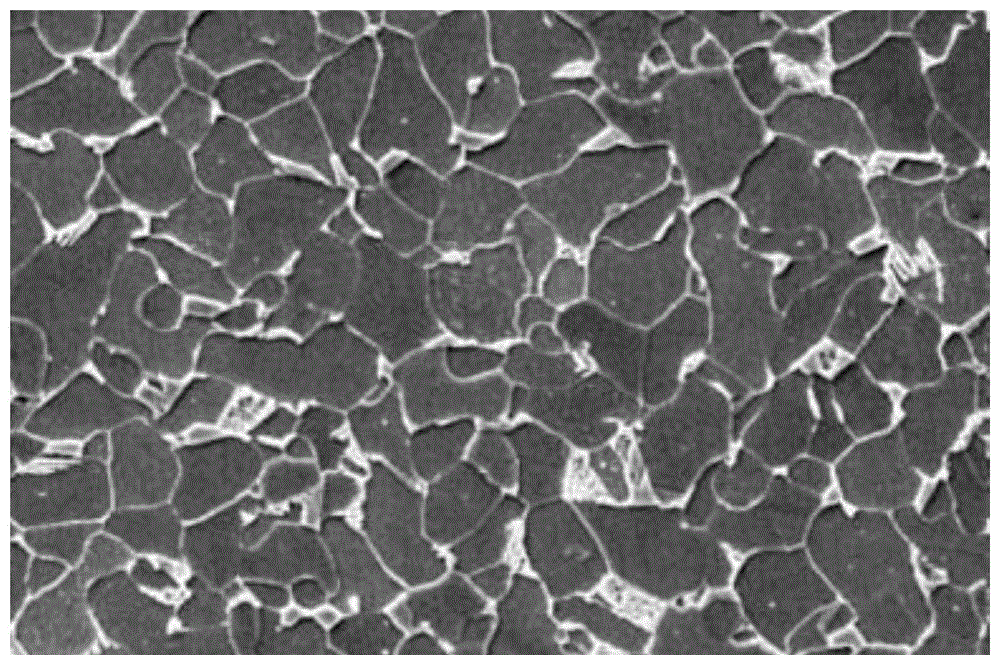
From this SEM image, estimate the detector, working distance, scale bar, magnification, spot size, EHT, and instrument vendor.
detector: SE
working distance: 10.1 mm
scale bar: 20000 nm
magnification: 2.4 K X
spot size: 4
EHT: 20 kV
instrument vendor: FEI